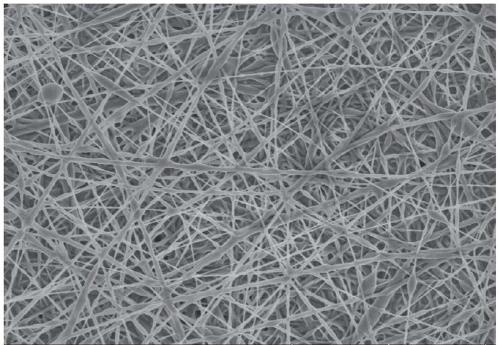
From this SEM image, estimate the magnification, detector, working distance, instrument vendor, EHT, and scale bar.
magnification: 5 K X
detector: SE(UL)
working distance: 9.3 mm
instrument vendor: Hitachi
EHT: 3 kV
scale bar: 10000 nm